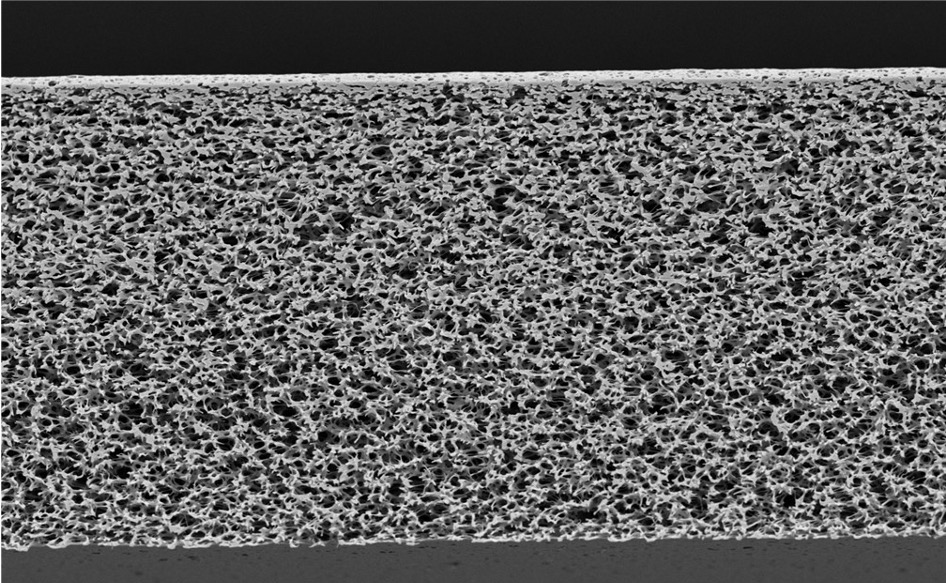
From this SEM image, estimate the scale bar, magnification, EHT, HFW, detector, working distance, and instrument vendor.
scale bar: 50000 nm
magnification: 0.6 K X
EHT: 10 kV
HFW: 212 µm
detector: Mix 50%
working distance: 5.865 mm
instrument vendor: Thermo